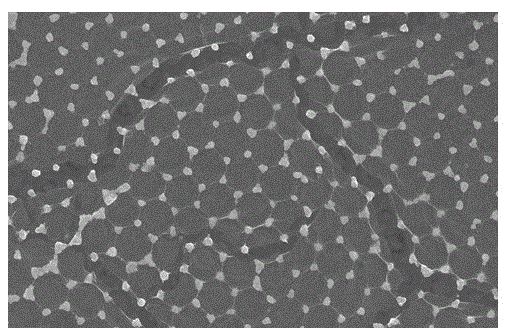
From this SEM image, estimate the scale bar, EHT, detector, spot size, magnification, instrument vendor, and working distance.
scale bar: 1000 nm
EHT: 12 kV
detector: TLD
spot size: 3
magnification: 20 K X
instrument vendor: FEI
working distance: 5.8 mm